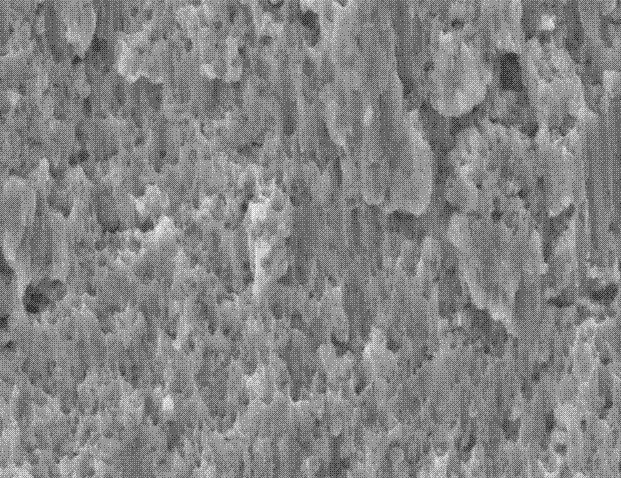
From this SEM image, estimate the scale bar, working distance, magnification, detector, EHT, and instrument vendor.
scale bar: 1000 nm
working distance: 7.5 mm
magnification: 20 K X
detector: SE2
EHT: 15 kV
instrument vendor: Zeiss